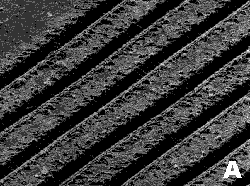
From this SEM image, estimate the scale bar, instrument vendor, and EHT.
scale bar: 100000 nm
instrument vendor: JEOL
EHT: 20 kV